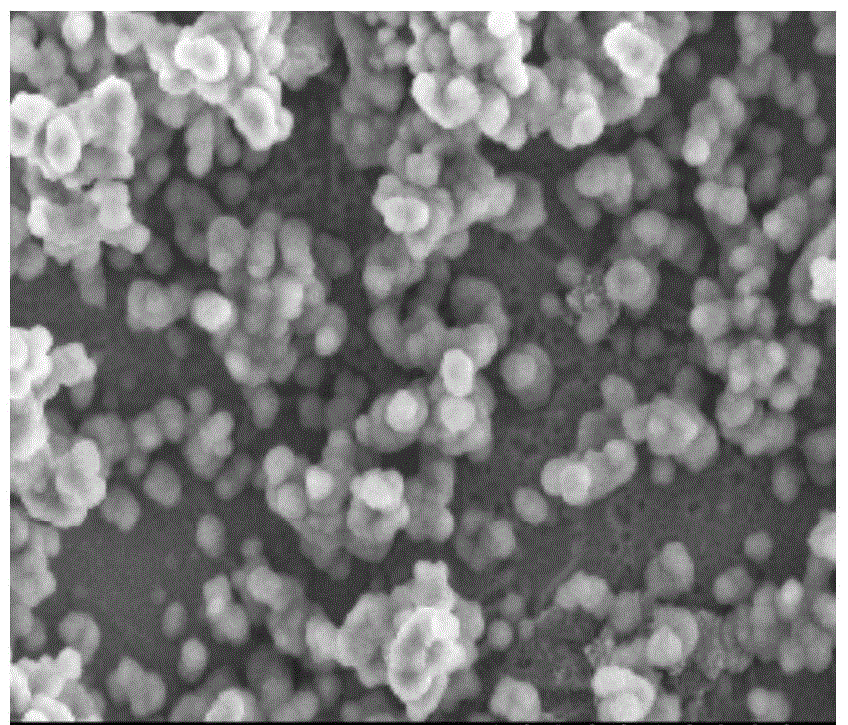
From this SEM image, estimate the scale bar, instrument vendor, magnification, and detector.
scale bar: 50000 nm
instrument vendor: Hitachi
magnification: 1 K X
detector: SE